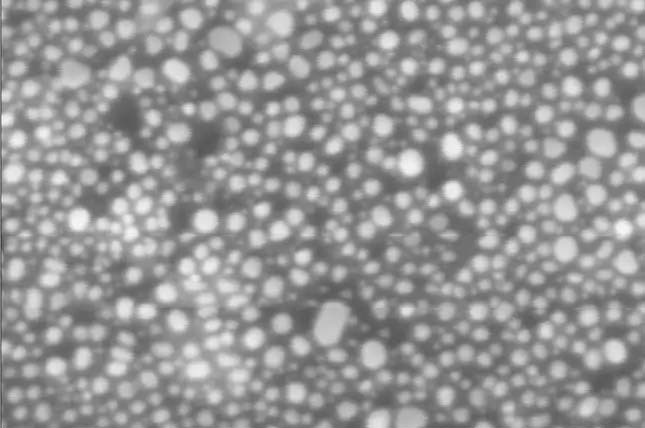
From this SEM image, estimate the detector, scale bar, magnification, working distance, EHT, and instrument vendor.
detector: InLens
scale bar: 30 nm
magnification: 500 K X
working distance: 3.6 mm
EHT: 5 kV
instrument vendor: Zeiss